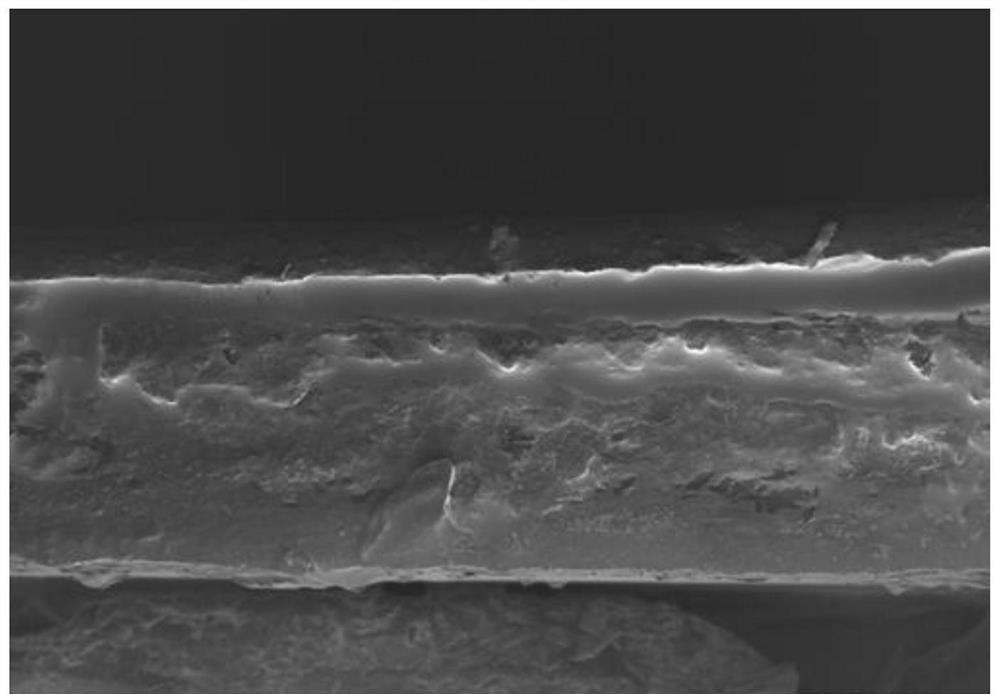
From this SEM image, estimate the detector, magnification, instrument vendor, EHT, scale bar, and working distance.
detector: InLens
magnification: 0.351 K X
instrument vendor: Zeiss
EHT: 1 kV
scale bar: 10000 nm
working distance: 7.2 mm